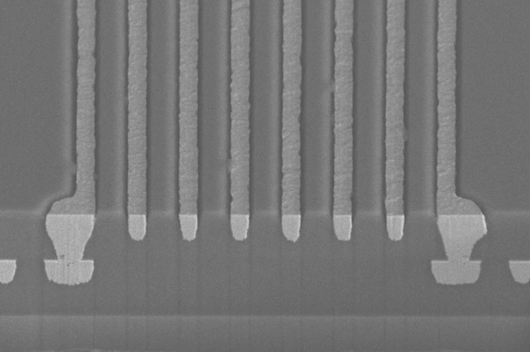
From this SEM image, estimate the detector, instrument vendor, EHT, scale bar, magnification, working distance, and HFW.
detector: TLD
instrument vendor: FEI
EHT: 15 kV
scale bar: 30000 nm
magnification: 2 K X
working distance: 4 mm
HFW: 104 µm